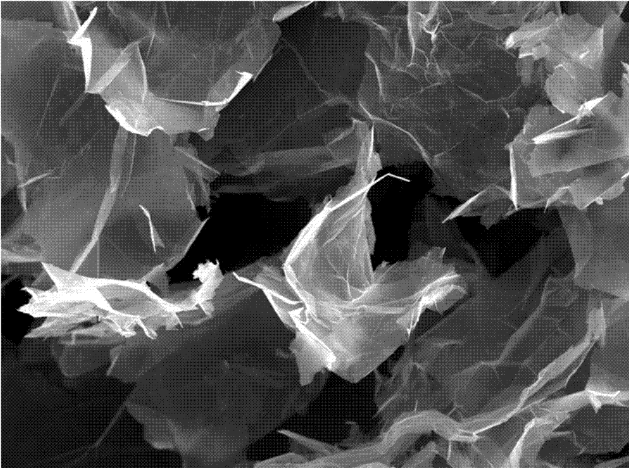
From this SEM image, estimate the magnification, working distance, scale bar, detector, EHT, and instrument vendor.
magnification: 5 K X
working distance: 6.6 mm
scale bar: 1000 nm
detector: InLens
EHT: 5 kV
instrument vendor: Zeiss